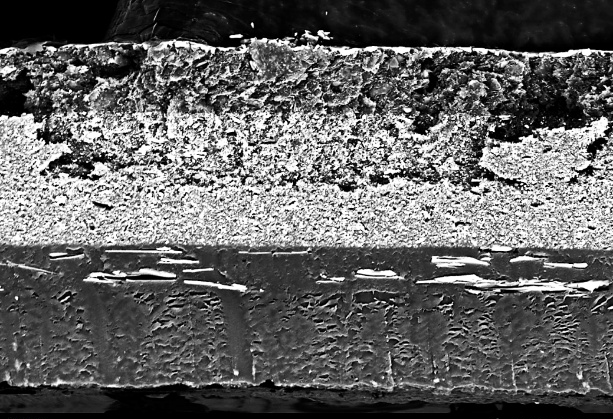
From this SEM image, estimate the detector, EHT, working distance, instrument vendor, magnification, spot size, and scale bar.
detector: BSE-COMPO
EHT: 15 kV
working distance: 13 mm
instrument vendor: other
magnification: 1 K X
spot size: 16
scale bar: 10000 nm